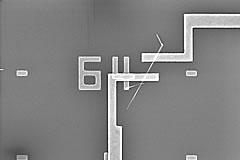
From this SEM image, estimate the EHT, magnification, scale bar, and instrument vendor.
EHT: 10 kV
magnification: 15 K X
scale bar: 3000 nm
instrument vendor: Hitachi